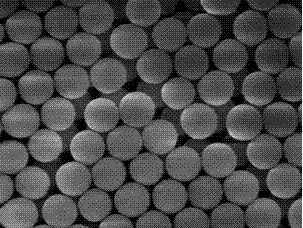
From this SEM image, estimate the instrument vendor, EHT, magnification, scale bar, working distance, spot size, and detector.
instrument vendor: FEI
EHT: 5 kV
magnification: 40 K X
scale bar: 500 nm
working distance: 4.9 mm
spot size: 3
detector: TLD